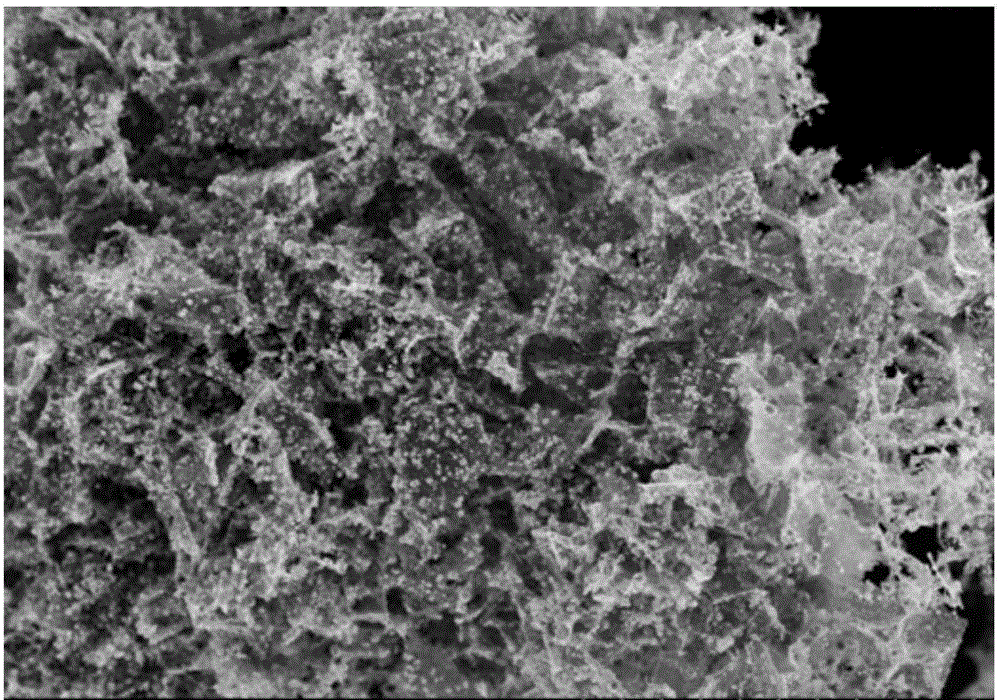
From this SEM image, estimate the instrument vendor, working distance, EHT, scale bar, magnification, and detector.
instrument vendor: Hitachi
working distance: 8.5 mm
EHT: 5 kV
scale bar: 10000 nm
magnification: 5 K X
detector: SE(M)